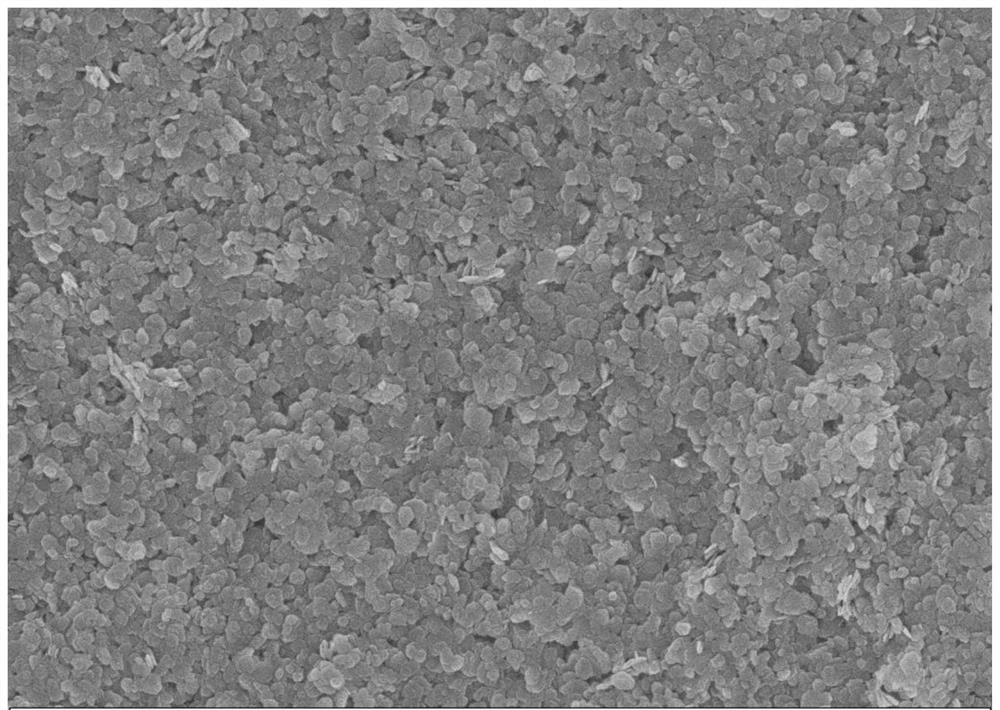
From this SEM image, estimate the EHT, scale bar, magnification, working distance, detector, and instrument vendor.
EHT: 5 kV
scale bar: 200 nm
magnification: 20 K X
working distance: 5.7 mm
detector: InLens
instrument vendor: Zeiss